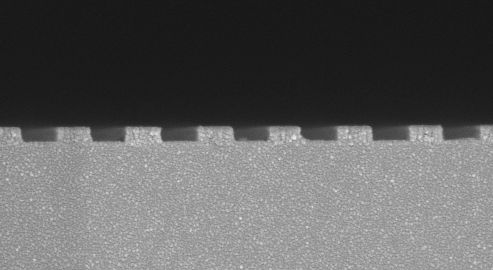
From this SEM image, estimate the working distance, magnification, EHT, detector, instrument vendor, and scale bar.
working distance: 6 mm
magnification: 100 K X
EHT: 5 kV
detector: InLens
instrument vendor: Zeiss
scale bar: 300 nm